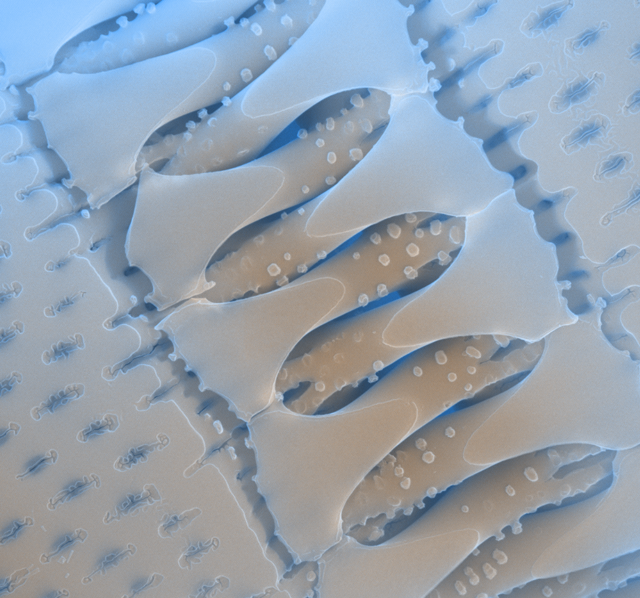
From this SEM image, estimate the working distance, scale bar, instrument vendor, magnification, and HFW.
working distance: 7.9 mm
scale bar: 5000 nm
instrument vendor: FEI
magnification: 13.796 K X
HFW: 10.8 µm